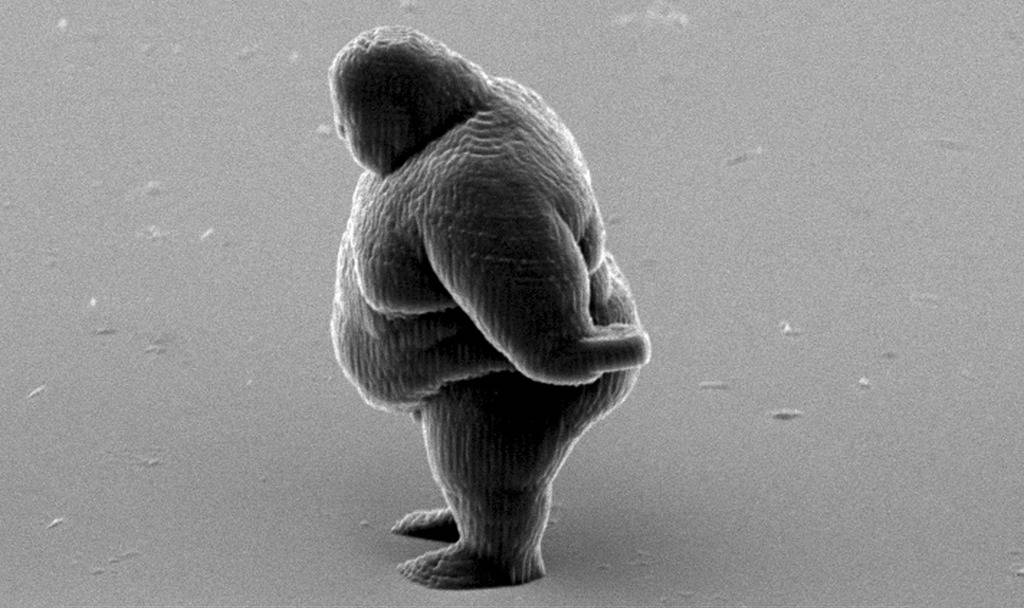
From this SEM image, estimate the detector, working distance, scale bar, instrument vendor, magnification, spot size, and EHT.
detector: SE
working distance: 33.5 mm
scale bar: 20000 nm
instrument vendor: FEI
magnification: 0.888 K X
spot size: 4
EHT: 12 kV